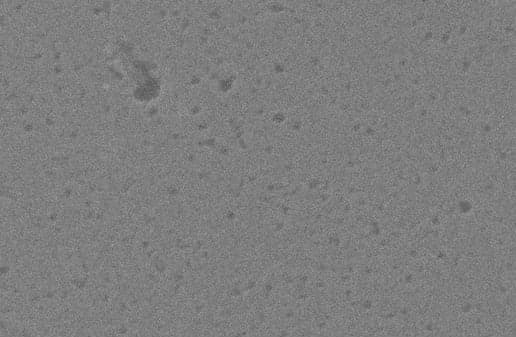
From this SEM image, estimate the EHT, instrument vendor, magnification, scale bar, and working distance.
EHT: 20 kV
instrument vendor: Zeiss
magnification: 54.69 K X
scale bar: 200 nm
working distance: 8.4 mm